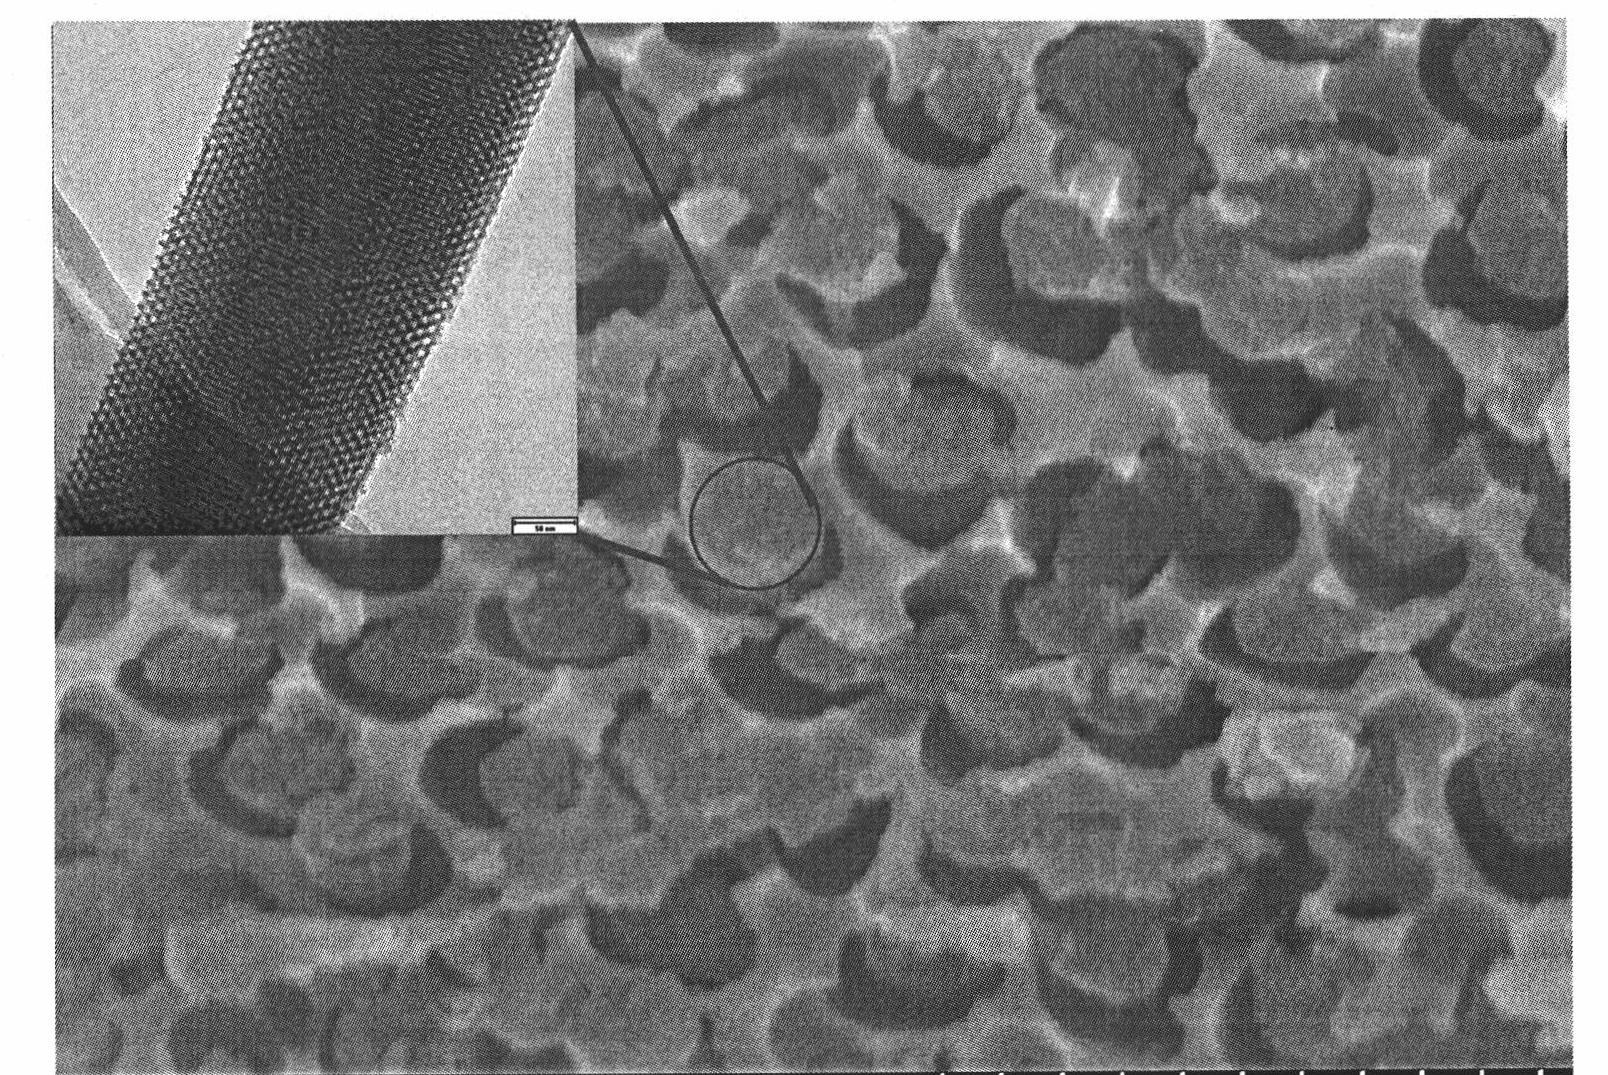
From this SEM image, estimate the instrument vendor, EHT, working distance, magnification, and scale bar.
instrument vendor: Hitachi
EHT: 20 kV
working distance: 11.5 mm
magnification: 50 K X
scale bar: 1000 nm